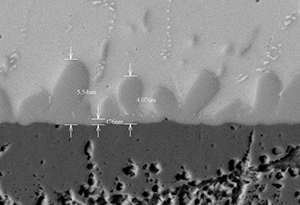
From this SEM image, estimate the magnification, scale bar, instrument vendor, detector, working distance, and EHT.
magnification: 6 K X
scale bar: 10000 nm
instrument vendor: Hitachi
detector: BSE3D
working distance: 8.9 mm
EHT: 15 kV